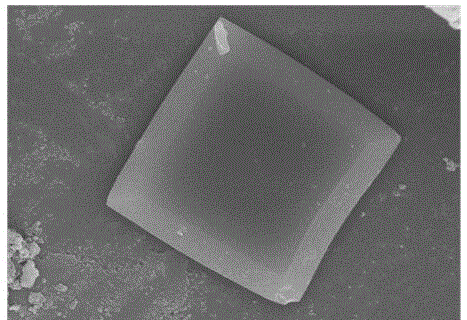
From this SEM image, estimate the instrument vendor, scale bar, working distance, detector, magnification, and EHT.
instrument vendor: Hitachi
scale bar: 5000 nm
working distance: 8.5 mm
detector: SE(U)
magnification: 11 K X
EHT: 10 kV